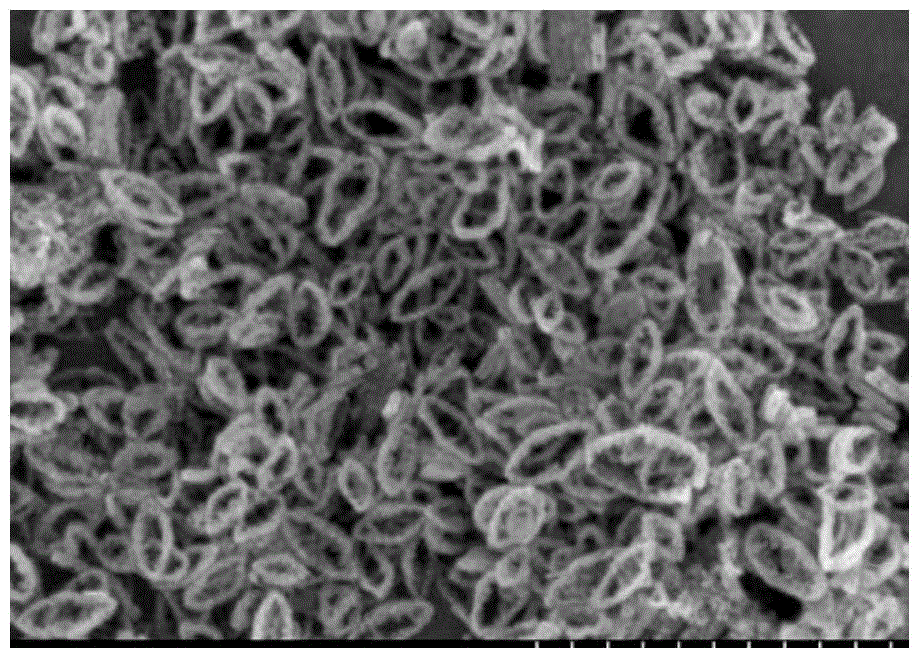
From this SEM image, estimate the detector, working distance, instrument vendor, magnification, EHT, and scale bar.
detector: SE(M)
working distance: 7.4 mm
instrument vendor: Hitachi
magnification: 50 K X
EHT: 5 kV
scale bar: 1000 nm